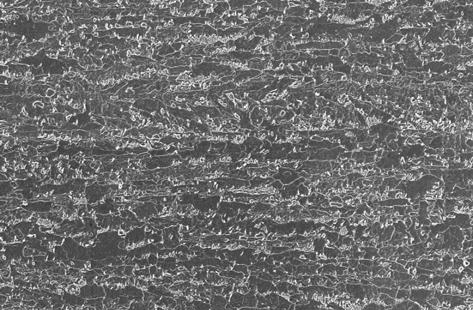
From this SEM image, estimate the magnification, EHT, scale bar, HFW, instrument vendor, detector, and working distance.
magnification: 5 K X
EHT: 5 kV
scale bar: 2000 nm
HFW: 59.59 µm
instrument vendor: Zeiss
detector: InLens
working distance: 2.5 mm